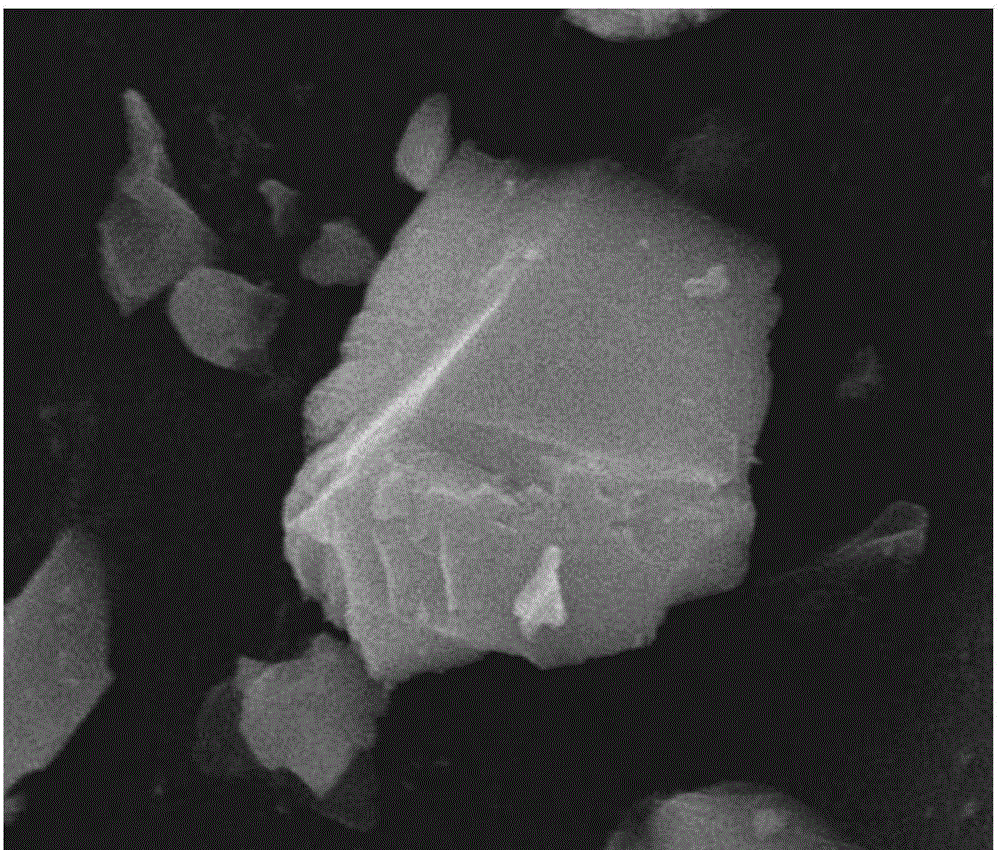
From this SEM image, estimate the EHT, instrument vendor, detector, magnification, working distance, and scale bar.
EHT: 20 kV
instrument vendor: FEI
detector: ETD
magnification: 12 K X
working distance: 12.7 mm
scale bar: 5000 nm